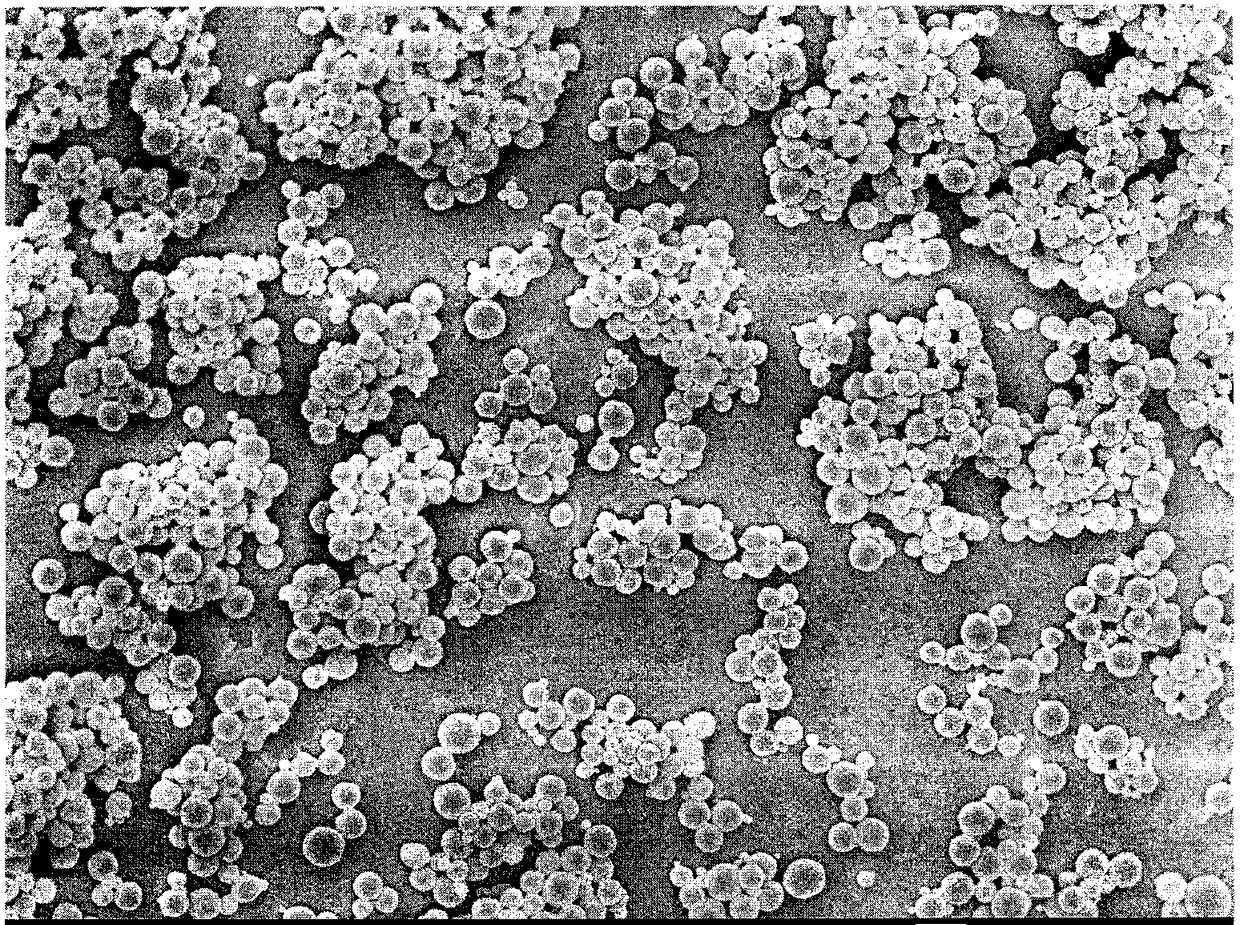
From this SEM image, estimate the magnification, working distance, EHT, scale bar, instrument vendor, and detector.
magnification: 5 K X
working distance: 7 mm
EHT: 5 kV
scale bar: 1000 nm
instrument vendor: JEOL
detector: SEI